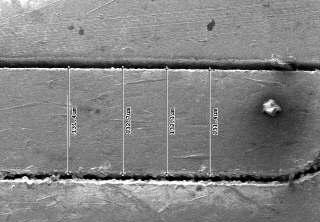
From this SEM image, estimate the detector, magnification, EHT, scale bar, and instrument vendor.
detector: SED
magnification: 0.3 K X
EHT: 5 kV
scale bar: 100000 nm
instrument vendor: other